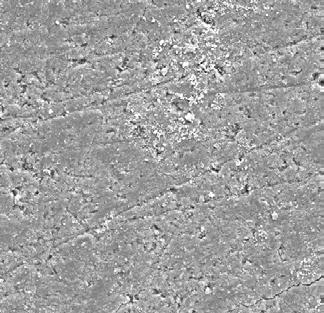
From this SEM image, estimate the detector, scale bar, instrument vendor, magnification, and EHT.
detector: SE Detector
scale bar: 100000 nm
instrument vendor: Tescan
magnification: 0.5 K X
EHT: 20 kV